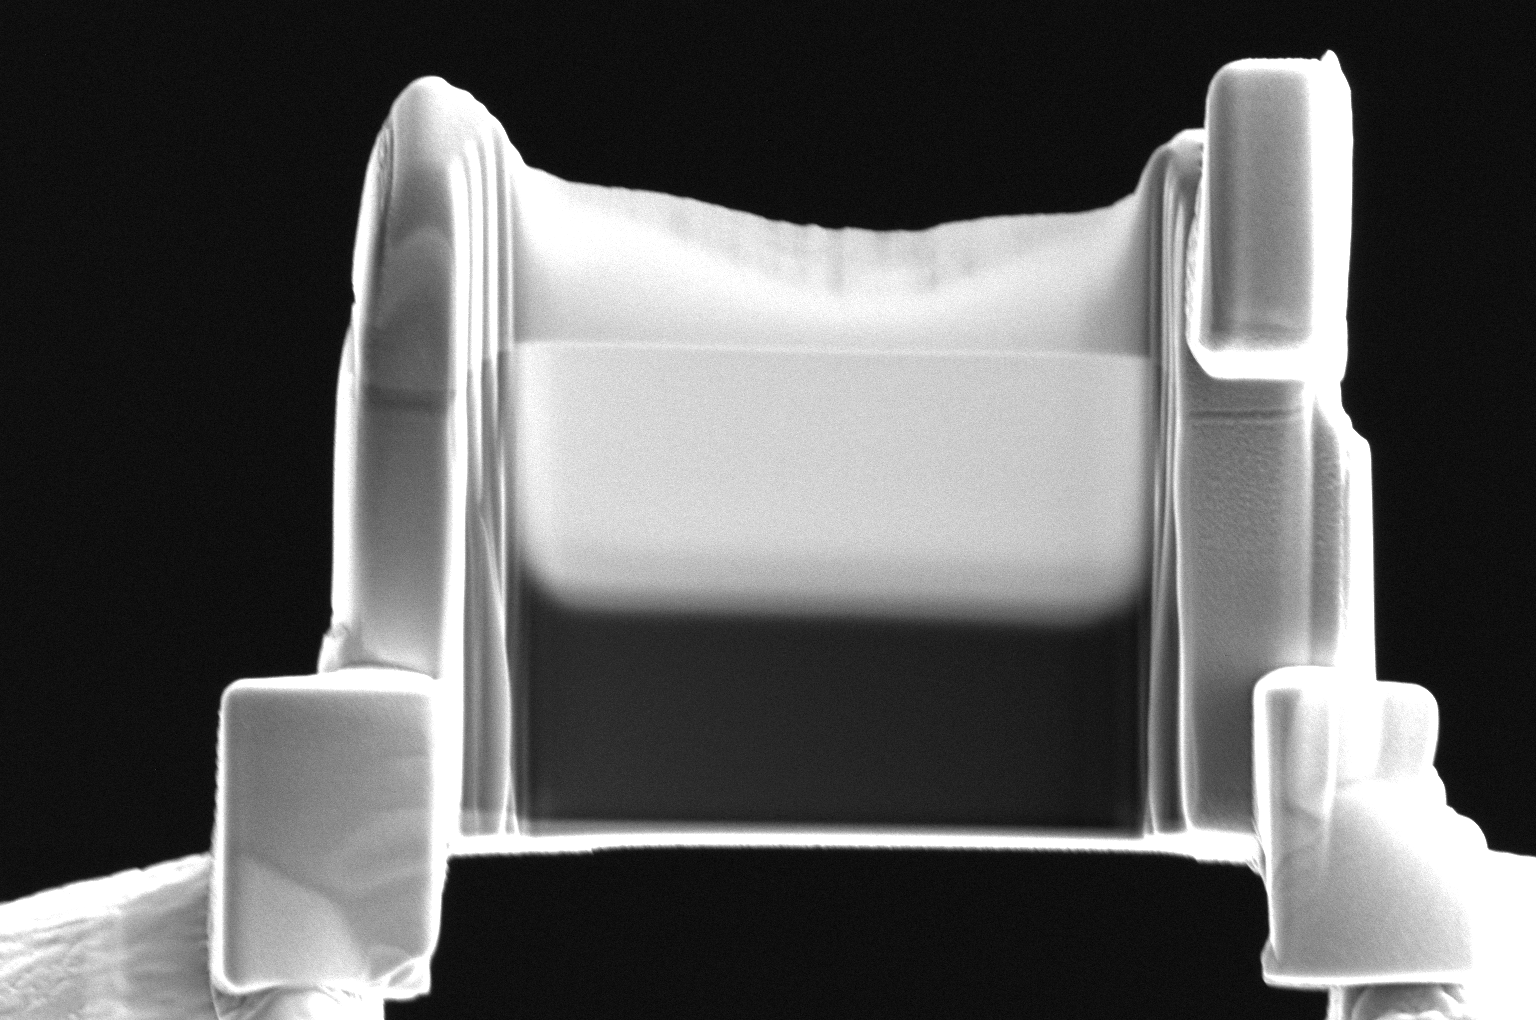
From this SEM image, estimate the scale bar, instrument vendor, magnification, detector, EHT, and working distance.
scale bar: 5000 nm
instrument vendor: Thermo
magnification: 24 K X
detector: ETD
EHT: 5 kV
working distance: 4 mm